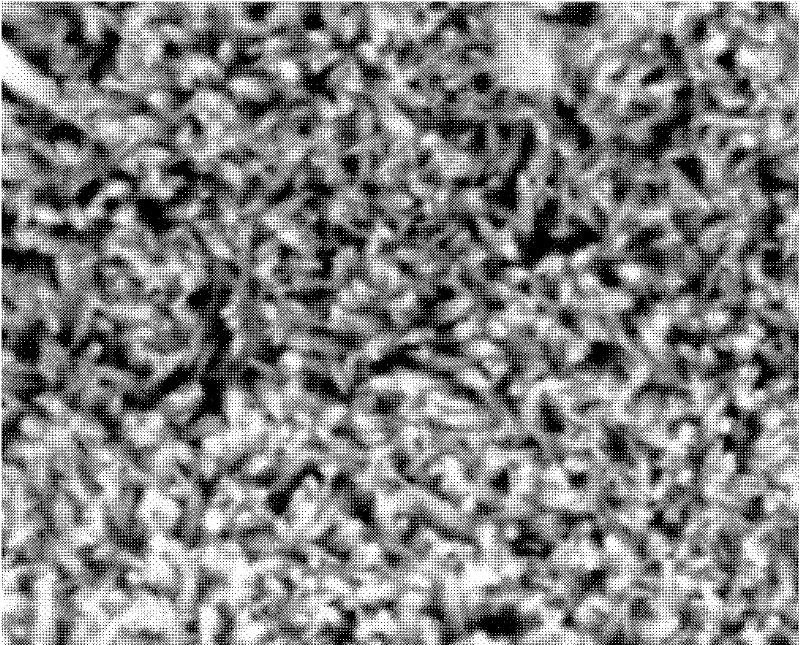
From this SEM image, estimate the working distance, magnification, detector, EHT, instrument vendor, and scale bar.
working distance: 14.1 mm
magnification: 20 K X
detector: ETD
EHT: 25 kV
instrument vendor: FEI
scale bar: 3000 nm